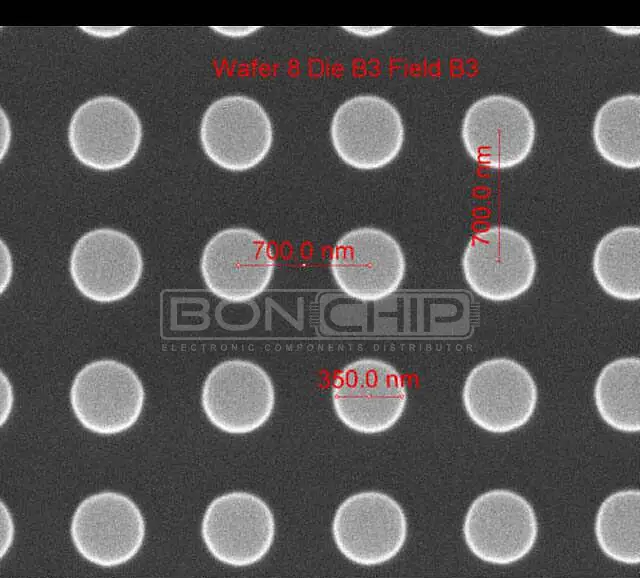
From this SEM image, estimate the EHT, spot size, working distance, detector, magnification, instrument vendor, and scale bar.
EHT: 10 kV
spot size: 1.5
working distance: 10.3 mm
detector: ETD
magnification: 75 K X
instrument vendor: FEI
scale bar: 1000 nm